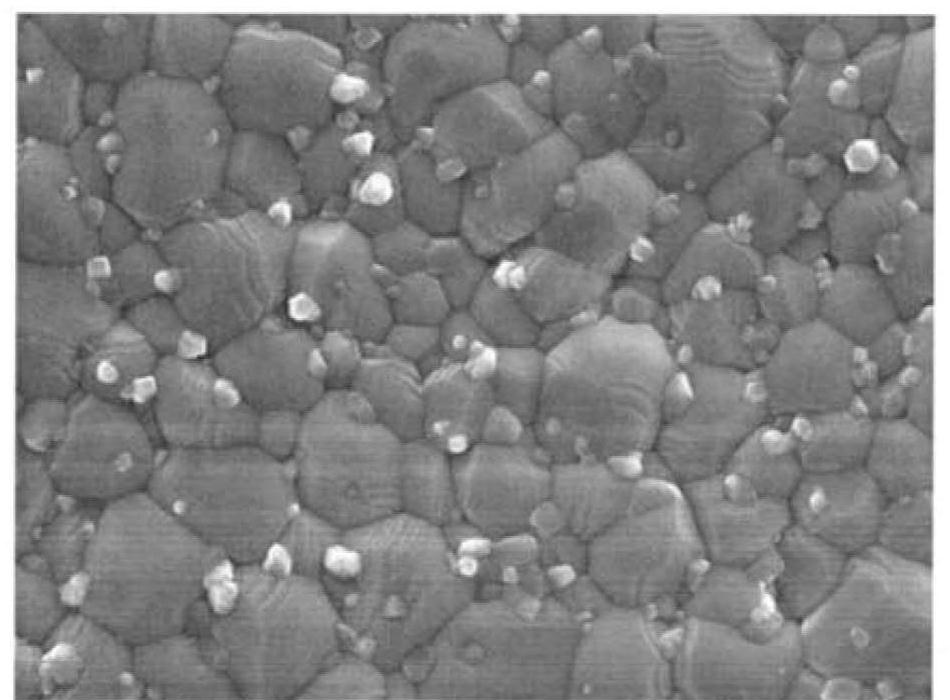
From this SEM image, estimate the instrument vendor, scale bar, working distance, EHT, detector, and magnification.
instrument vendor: JEOL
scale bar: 1000 nm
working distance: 9 mm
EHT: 10 kV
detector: SEI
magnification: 10 K X